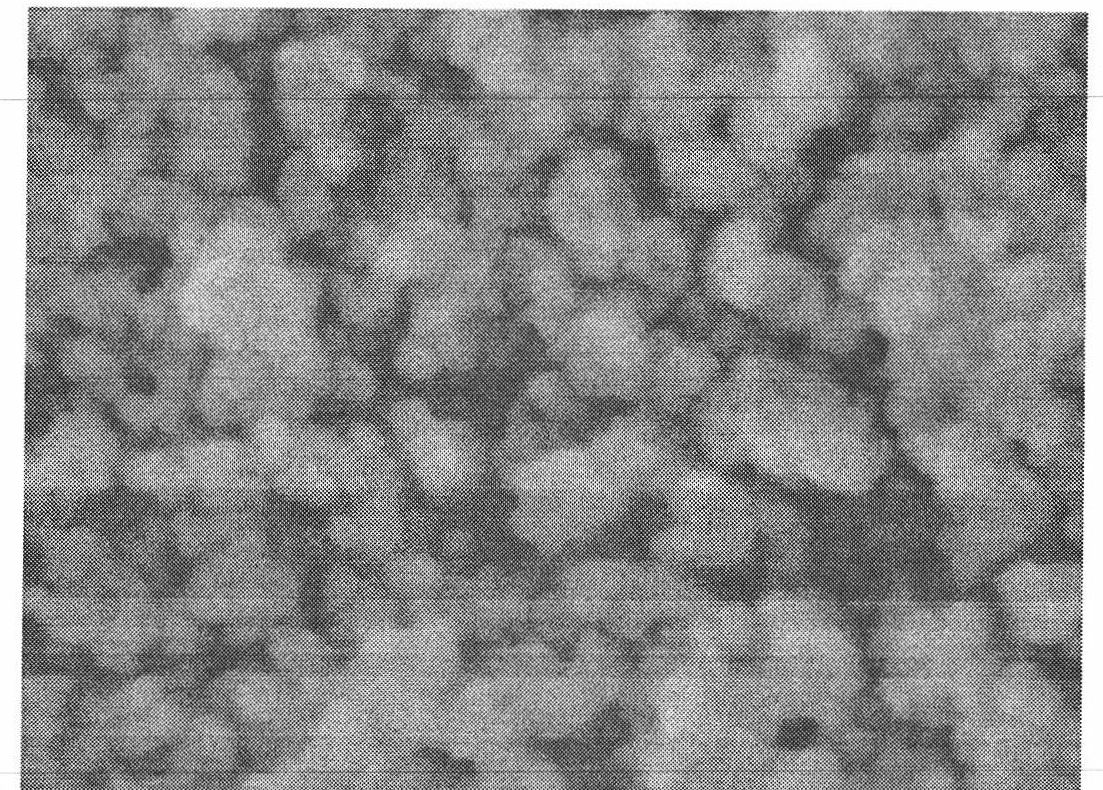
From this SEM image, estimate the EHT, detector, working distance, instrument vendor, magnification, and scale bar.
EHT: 5 kV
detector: SEI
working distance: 8.5 mm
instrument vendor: JEOL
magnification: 100 K X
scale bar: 100 nm